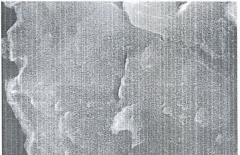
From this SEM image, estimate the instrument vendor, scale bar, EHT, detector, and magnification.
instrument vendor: JEOL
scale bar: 1000 nm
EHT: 20 kV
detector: SEI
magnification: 14 K X